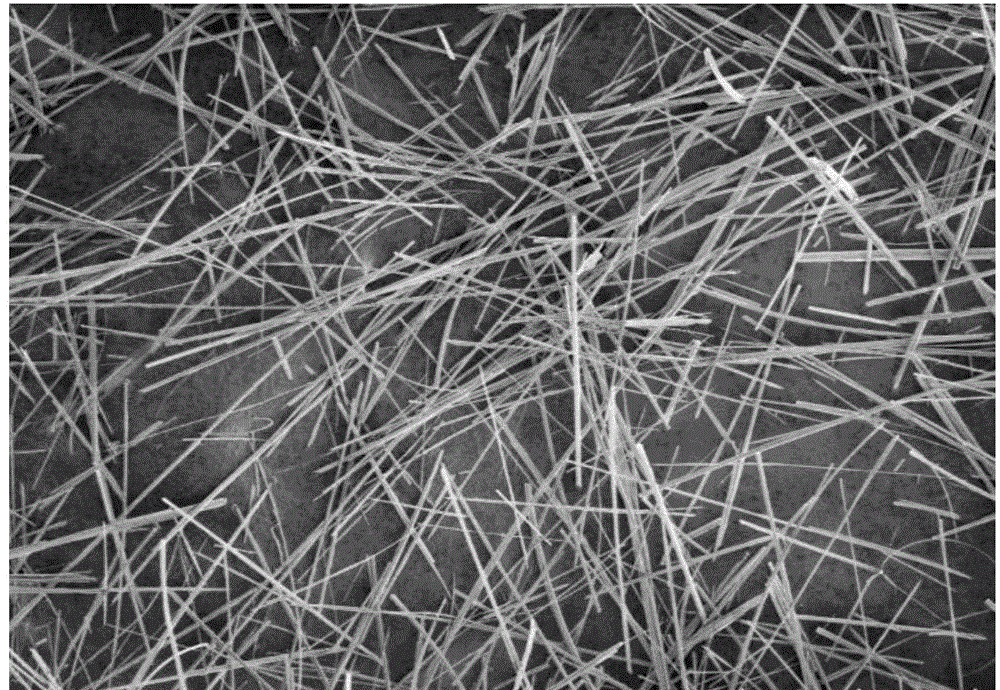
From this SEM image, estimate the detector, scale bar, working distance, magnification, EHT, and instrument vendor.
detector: SE(UL)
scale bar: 100000 nm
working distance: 11.1 mm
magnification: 0.3 K X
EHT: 1 kV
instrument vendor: Hitachi